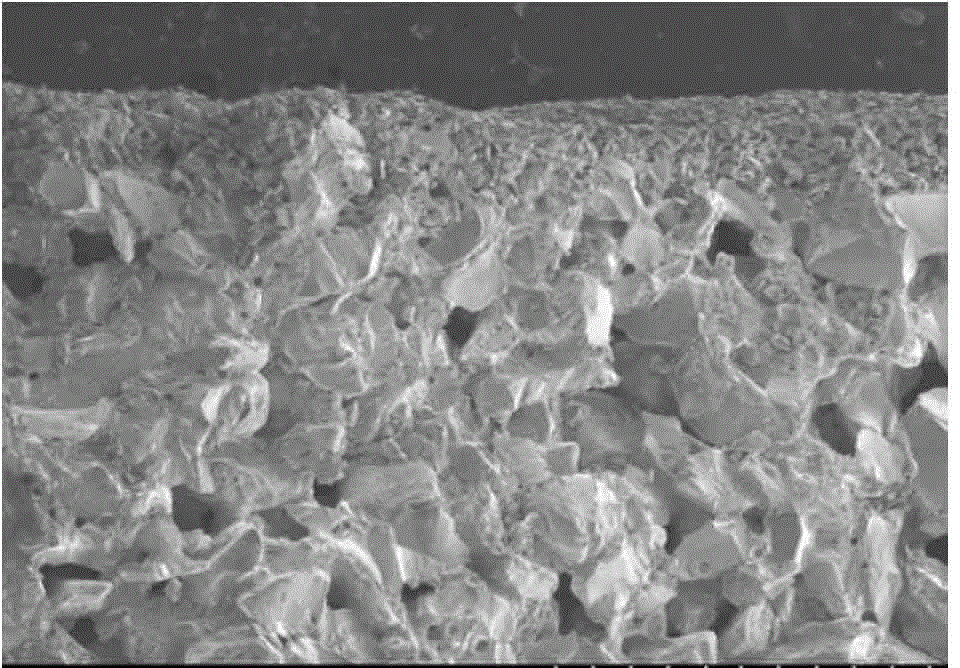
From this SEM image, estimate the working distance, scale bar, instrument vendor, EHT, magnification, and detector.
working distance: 8.2 mm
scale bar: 1000000 nm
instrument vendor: Hitachi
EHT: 5 kV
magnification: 0.05 K X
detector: SE(M)